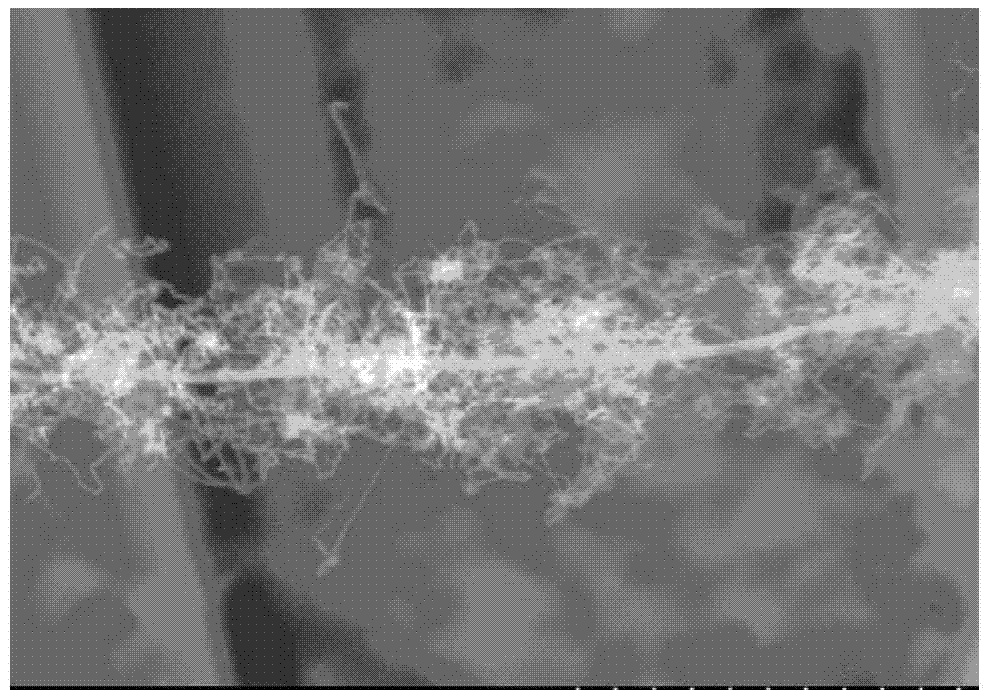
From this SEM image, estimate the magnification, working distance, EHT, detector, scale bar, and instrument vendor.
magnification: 5 K X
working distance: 11.7 mm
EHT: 15 kV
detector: SE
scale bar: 10000 nm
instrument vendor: Hitachi